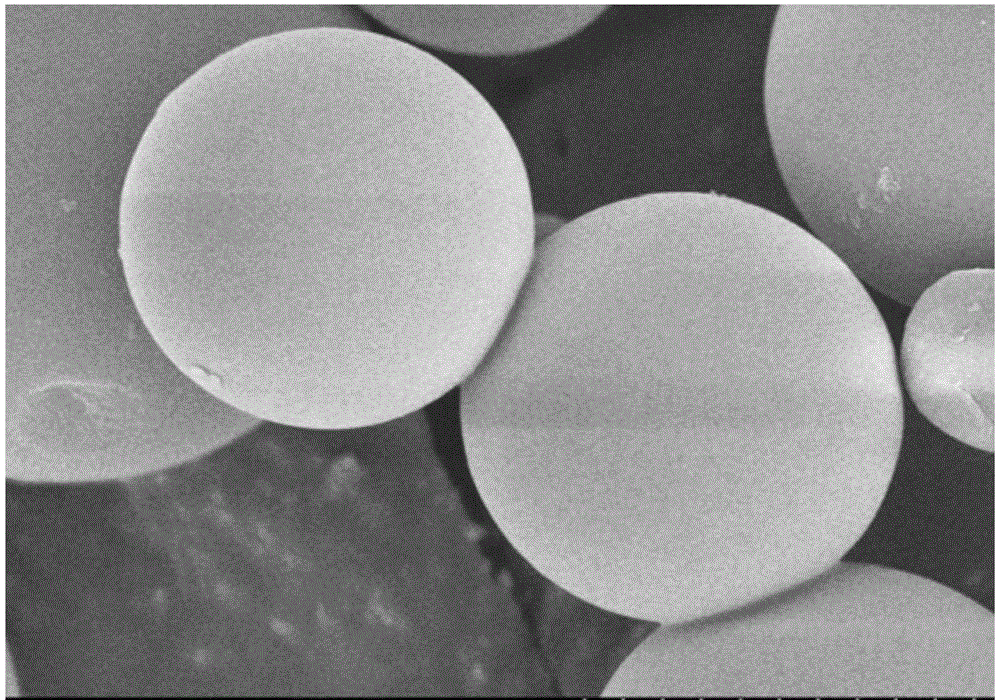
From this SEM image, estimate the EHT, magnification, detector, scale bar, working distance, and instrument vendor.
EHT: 5 kV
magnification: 10 K X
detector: SE(M,LA0)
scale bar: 5000 nm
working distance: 8.6 mm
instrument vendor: Hitachi